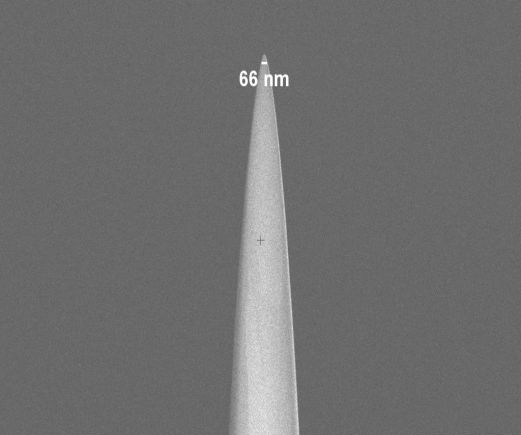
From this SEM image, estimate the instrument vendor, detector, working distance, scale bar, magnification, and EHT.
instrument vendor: Zeiss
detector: InLens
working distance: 4.7 mm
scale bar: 2000 nm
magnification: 20 K X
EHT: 2 kV